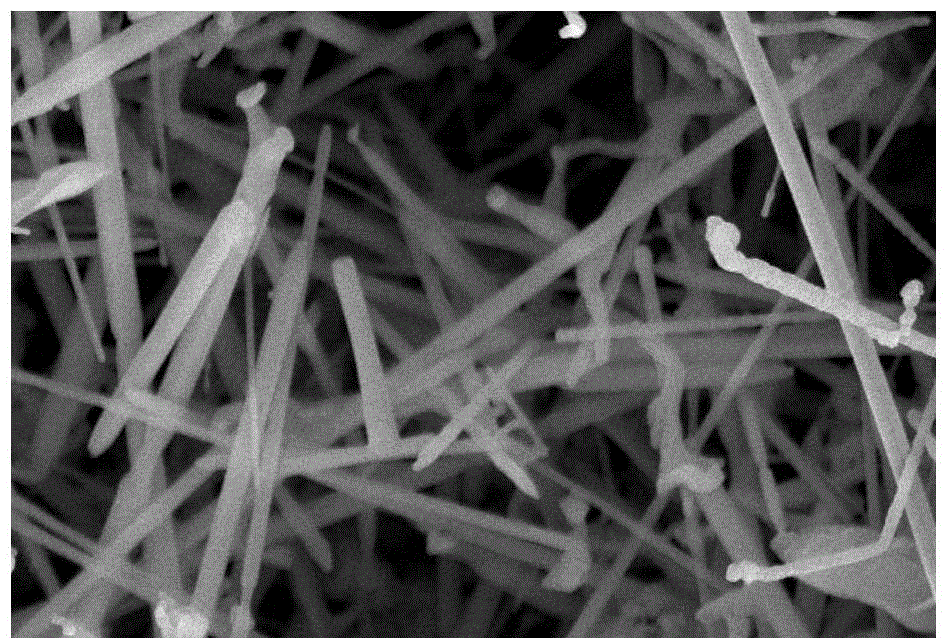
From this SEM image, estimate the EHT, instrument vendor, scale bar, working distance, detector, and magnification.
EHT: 10 kV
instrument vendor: Hitachi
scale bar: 2000 nm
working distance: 15.5 mm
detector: SE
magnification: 15 K X